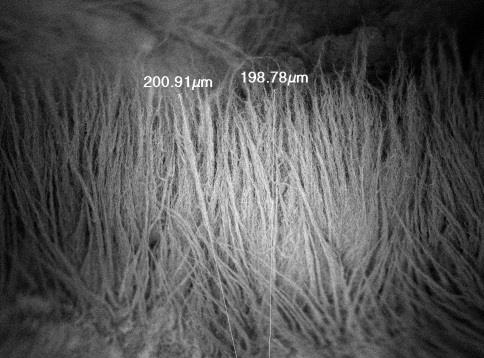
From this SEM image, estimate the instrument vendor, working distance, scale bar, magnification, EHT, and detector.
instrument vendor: JEOL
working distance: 5.9 mm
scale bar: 10000 nm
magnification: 0.35 K X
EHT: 2 kV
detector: SEI